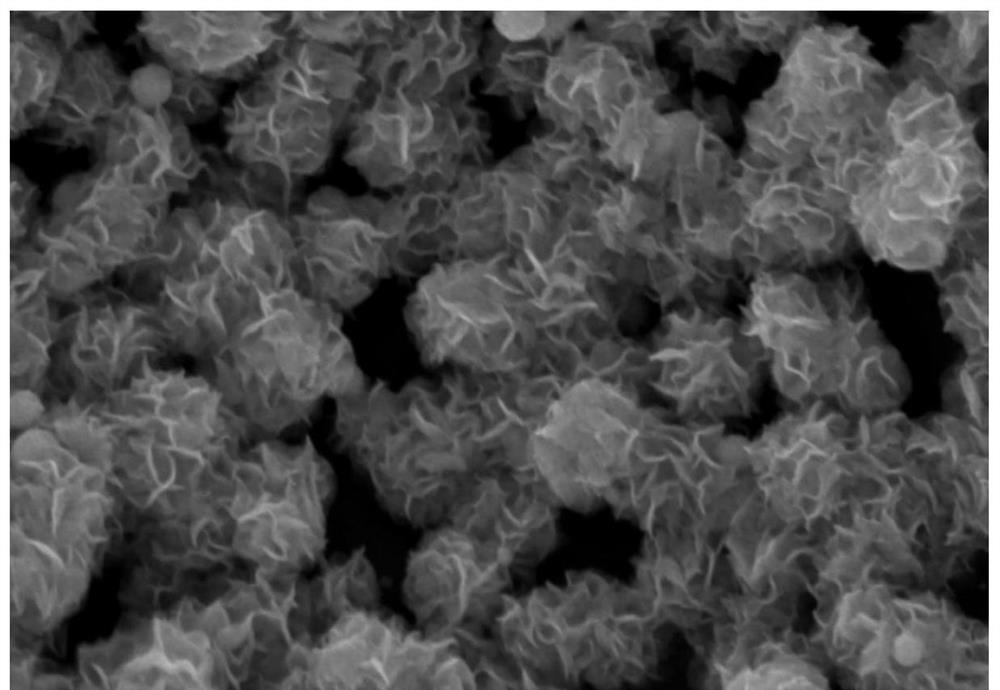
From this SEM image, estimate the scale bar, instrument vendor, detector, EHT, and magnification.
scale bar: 400 nm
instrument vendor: Hitachi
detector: SE(UL)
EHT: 5 kV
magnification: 200 K X